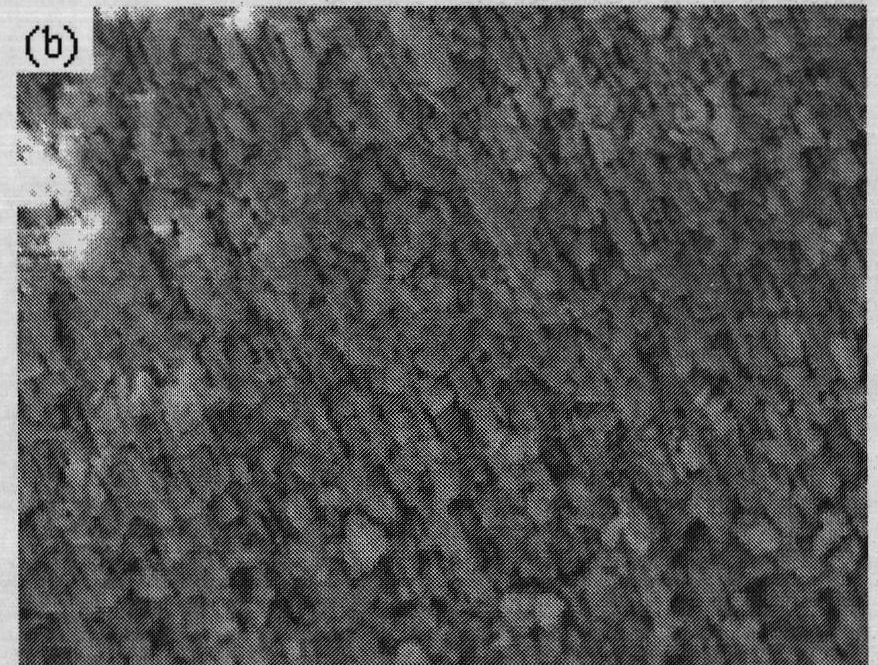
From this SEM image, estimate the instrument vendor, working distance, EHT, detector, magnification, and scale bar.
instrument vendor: JEOL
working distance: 9 mm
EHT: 15 kV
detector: SEI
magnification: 5 K X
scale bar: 1000 nm